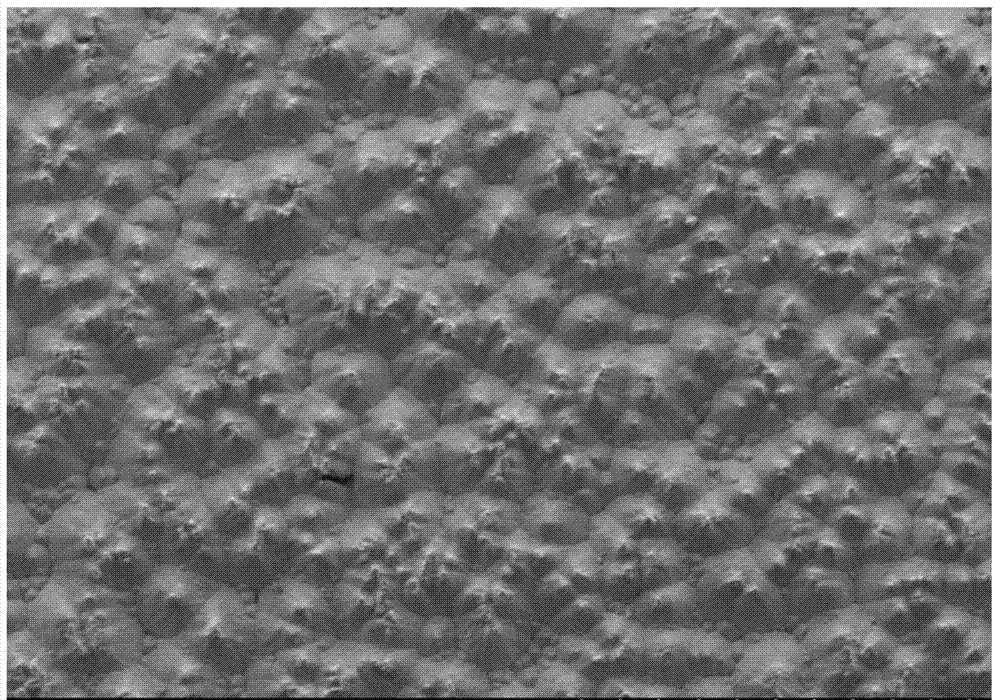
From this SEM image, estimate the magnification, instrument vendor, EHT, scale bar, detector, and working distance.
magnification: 0.05 K X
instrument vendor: Hitachi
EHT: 15 kV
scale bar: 1000000 nm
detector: SE(M)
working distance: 15 mm